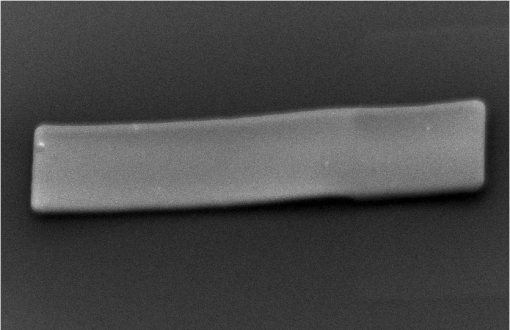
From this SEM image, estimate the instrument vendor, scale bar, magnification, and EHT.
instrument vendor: JEOL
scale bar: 20000 nm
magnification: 0.6 K X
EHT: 15 kV